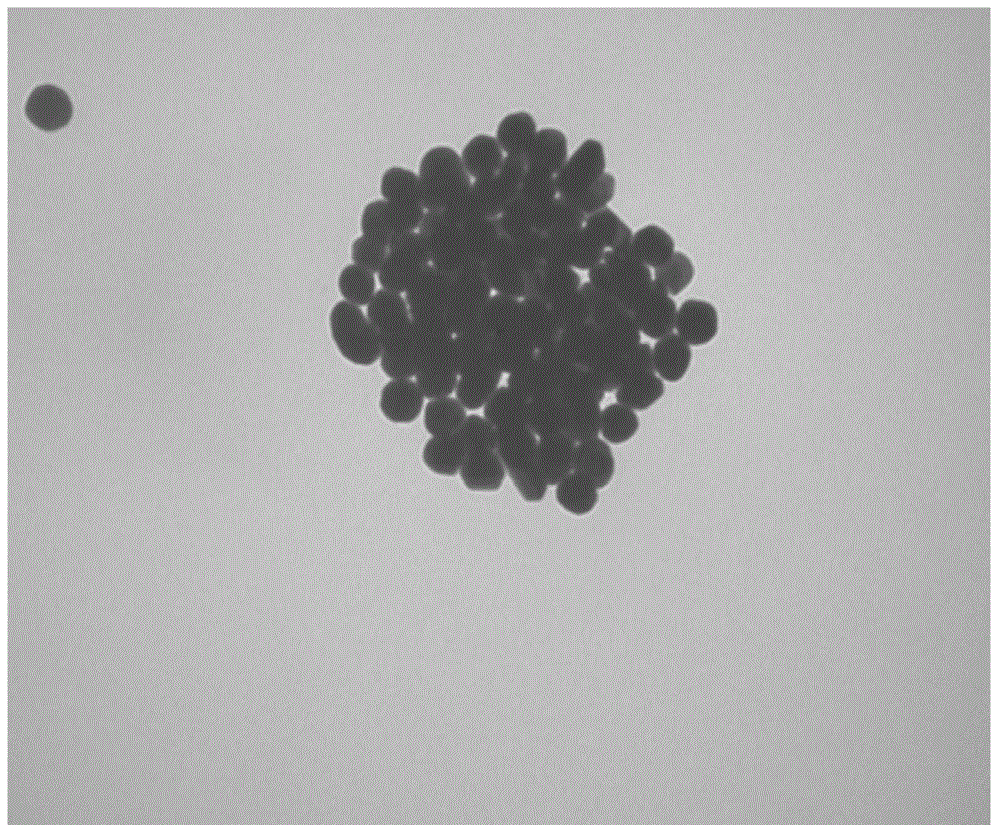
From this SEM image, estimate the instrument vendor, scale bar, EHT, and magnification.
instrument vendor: Hitachi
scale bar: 200 nm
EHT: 100 kV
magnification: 100 K X